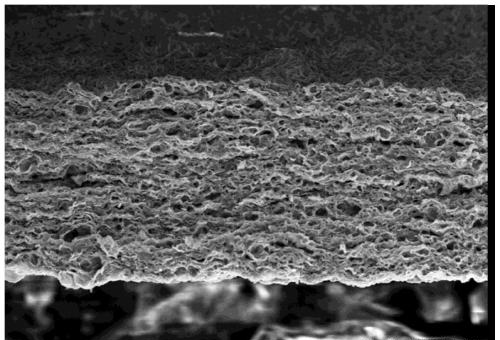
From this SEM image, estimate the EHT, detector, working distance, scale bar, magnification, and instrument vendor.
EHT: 15 kV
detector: SE(U)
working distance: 18.5 mm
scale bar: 50000 nm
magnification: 0.6 K X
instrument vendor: Hitachi